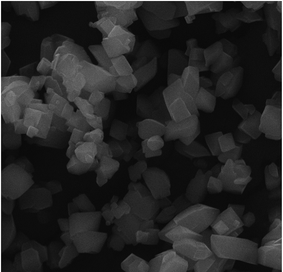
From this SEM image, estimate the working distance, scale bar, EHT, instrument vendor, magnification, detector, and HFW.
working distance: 10.51 mm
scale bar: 5000 nm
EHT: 20 kV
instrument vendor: Tescan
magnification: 7 K X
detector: SE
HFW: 29.7 µm